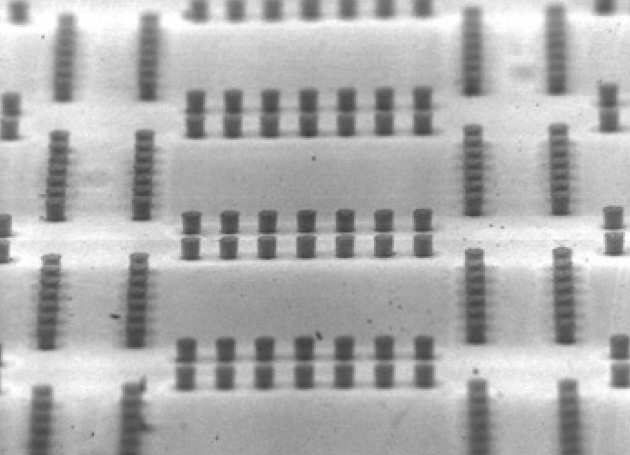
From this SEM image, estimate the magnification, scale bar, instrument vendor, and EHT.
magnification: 0.05 K X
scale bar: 600000 nm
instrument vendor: JEOL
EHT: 6 kV